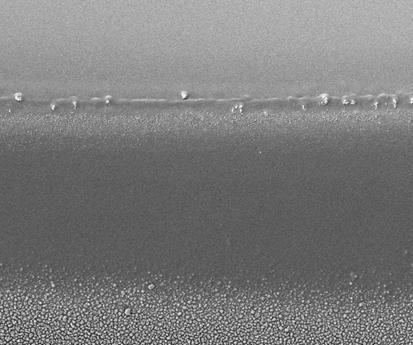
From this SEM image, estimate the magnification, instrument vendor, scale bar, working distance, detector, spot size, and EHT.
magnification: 10 K X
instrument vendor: FEI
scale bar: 10000 nm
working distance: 5.1 mm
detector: ETD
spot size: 3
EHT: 5 kV